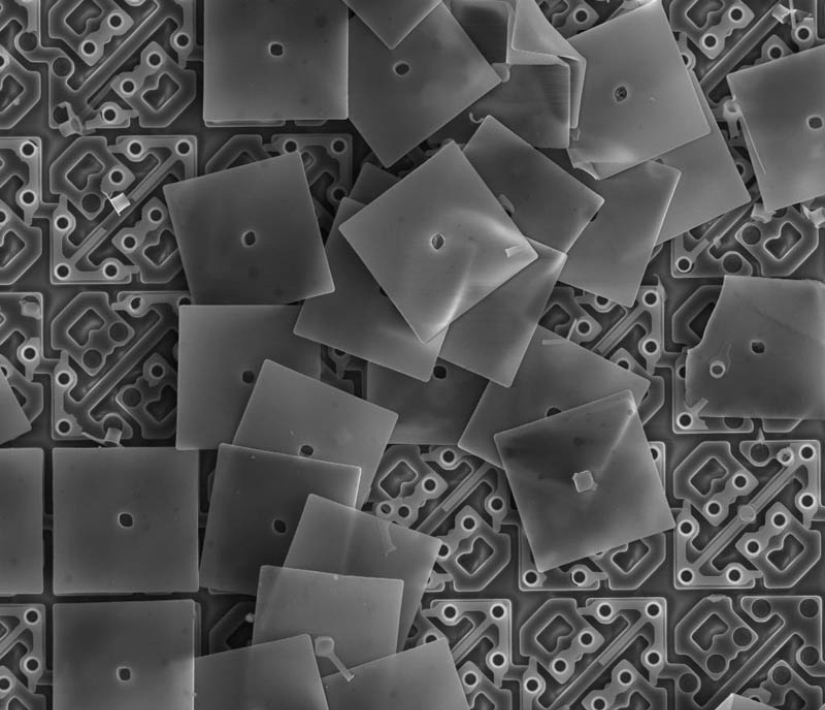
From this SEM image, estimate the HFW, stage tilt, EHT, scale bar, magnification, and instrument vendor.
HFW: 73.1 µm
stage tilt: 0°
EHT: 10 kV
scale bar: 30000 nm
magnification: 3.5 K X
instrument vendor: FEI